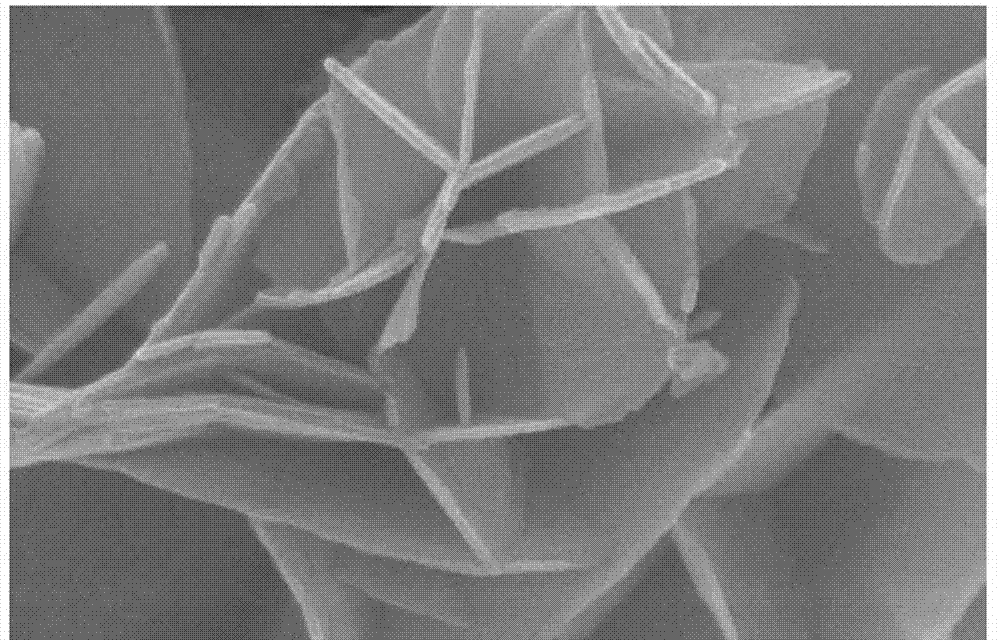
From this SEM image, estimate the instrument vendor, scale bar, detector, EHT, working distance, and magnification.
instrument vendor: Hitachi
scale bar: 500 nm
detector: SE(M)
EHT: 15 kV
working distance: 8.7 mm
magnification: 100 K X